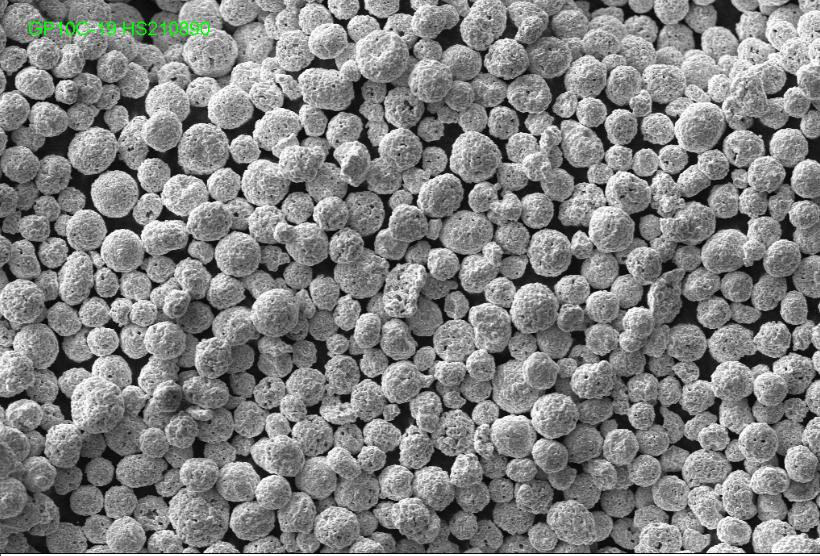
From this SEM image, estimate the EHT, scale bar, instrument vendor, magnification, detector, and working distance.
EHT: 20 kV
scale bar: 20000 nm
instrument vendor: Zeiss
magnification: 0.3 K X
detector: SE1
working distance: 11 mm